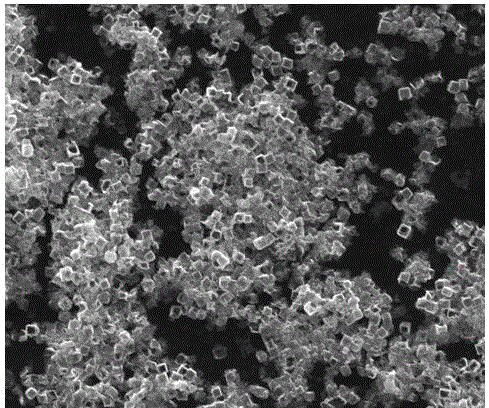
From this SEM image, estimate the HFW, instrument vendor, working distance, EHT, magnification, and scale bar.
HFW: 29.8 µm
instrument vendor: FEI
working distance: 12.3 mm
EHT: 25 kV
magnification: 10 K X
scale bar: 10000 nm